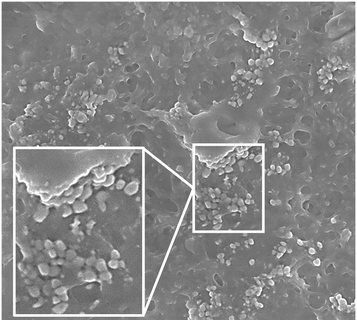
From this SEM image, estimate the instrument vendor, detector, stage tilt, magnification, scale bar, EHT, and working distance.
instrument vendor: Zeiss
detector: InLens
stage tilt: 0°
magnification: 60 K X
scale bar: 300 nm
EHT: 4 kV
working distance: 5 mm